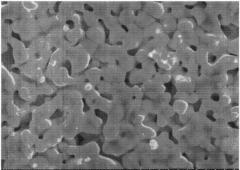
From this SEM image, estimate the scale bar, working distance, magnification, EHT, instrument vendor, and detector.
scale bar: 5000 nm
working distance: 9.7 mm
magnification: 10 K X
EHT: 15 kV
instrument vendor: Hitachi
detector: SE